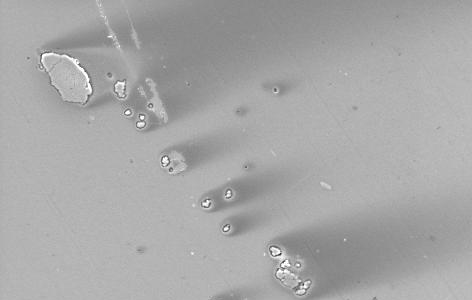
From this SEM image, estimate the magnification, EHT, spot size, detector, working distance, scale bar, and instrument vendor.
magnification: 1 K X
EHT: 20 kV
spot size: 5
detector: SE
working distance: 9.9 mm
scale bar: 50000 nm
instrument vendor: FEI